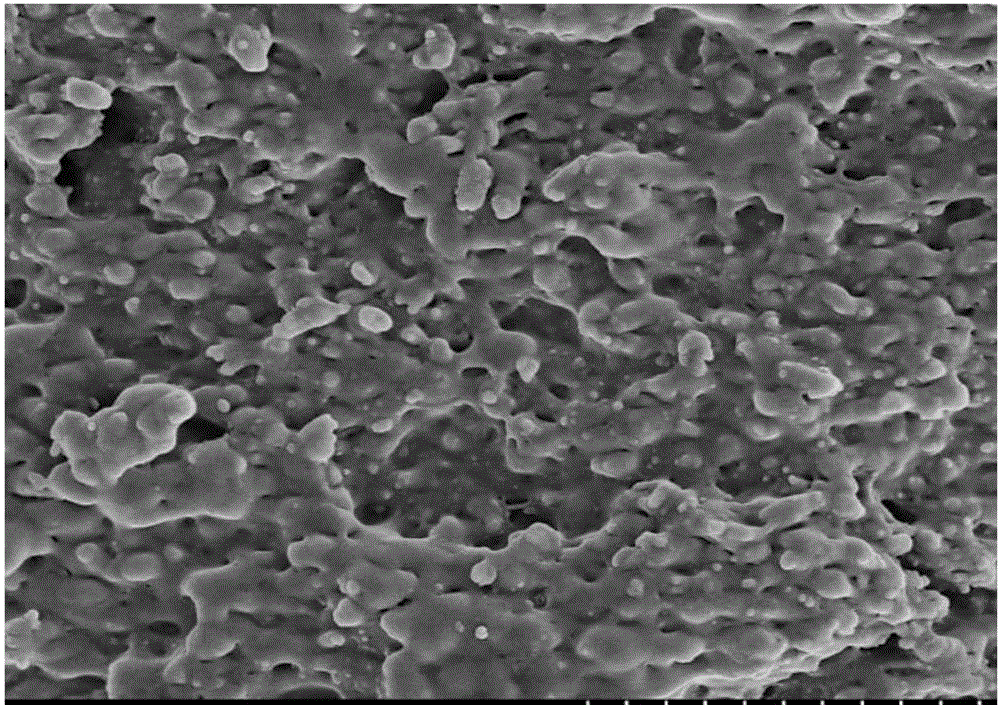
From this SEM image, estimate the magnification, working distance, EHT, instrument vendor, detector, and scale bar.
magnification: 10 K X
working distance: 9.2 mm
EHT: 5 kV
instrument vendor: Hitachi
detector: SE(M)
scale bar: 5000 nm